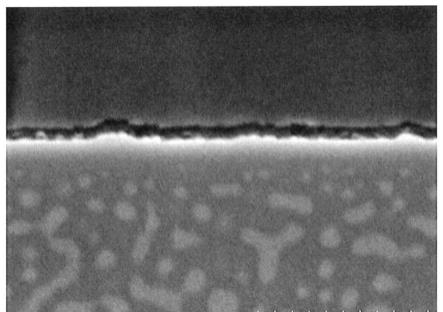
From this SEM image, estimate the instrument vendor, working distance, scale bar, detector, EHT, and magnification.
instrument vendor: Hitachi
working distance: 2.2 mm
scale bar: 500 nm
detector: SE(U,LA100)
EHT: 2 kV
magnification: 100 K X